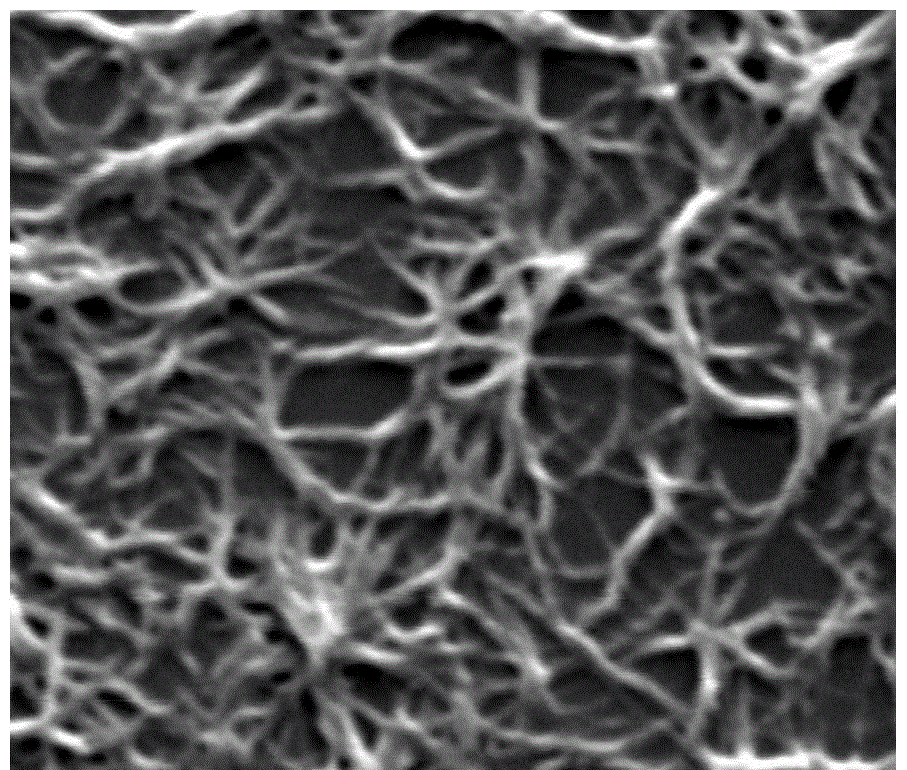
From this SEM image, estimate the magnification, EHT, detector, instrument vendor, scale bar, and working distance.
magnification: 20 K X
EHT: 20 kV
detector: ETD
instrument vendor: FEI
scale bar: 2000 nm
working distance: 10.8 mm